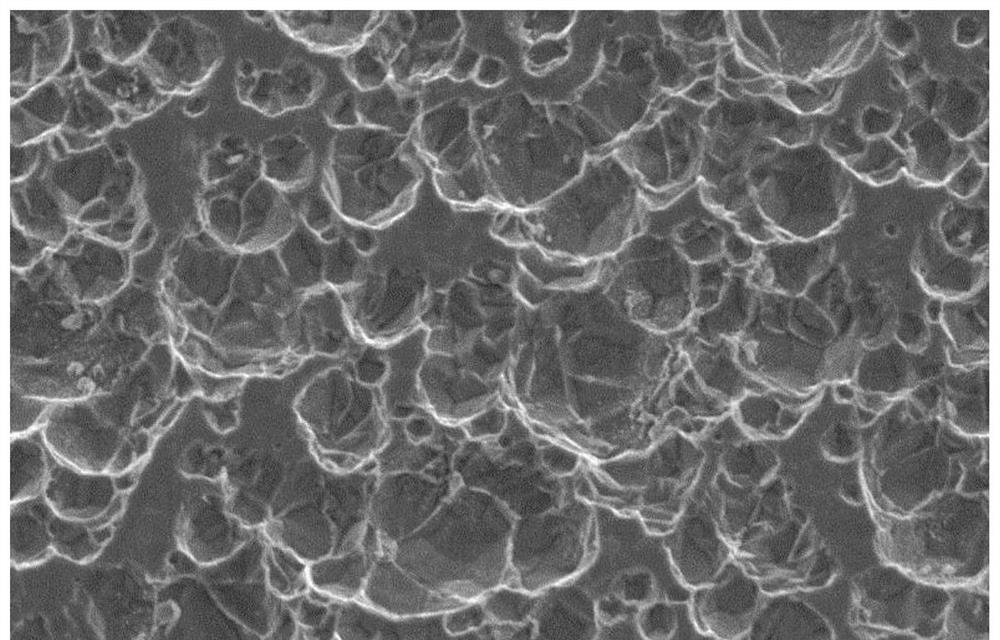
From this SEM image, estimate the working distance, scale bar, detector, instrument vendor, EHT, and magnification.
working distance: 9.7 mm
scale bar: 10000 nm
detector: SE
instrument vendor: Hitachi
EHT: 10 kV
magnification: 3 K X